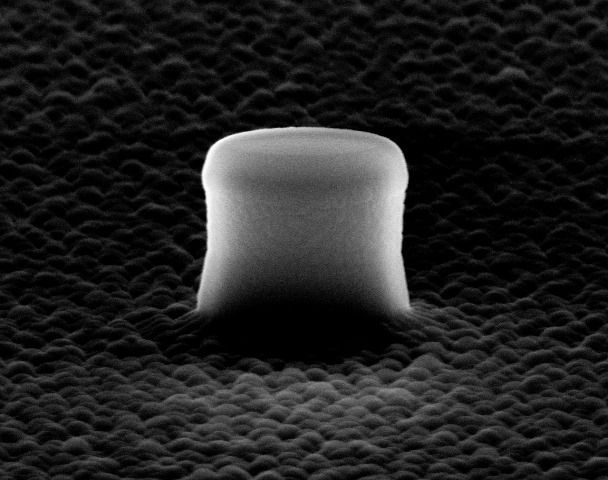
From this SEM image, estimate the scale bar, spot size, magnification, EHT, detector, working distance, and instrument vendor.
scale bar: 1000 nm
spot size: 3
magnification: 27.382 K X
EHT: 20 kV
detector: SE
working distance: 7.2 mm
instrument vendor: FEI